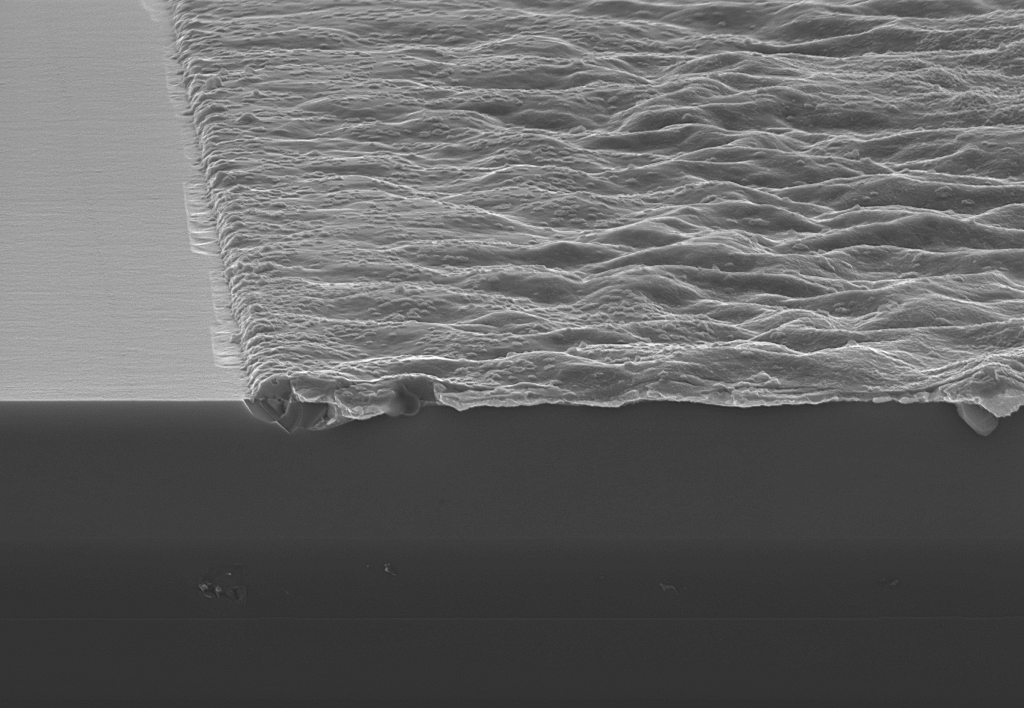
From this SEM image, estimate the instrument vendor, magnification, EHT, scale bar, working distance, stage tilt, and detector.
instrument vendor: Zeiss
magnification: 50 K X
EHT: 7 kV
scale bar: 200 nm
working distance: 3 mm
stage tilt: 7°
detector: InLens